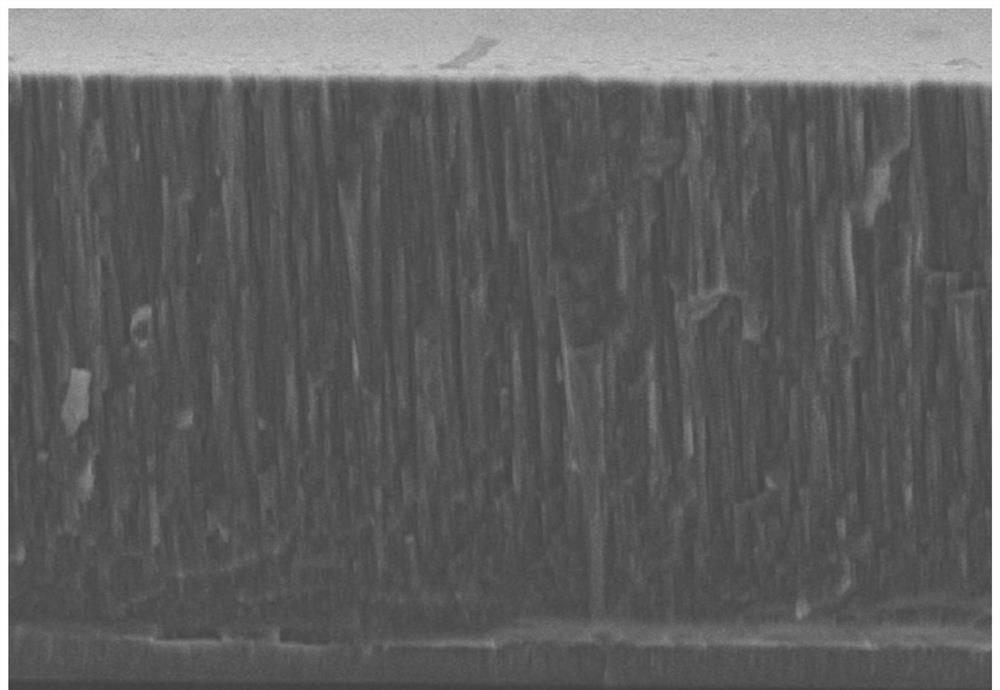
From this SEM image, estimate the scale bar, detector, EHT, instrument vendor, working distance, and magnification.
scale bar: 10000 nm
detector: SE(M)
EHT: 10 kV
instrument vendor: Hitachi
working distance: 7.1 mm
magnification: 4 K X